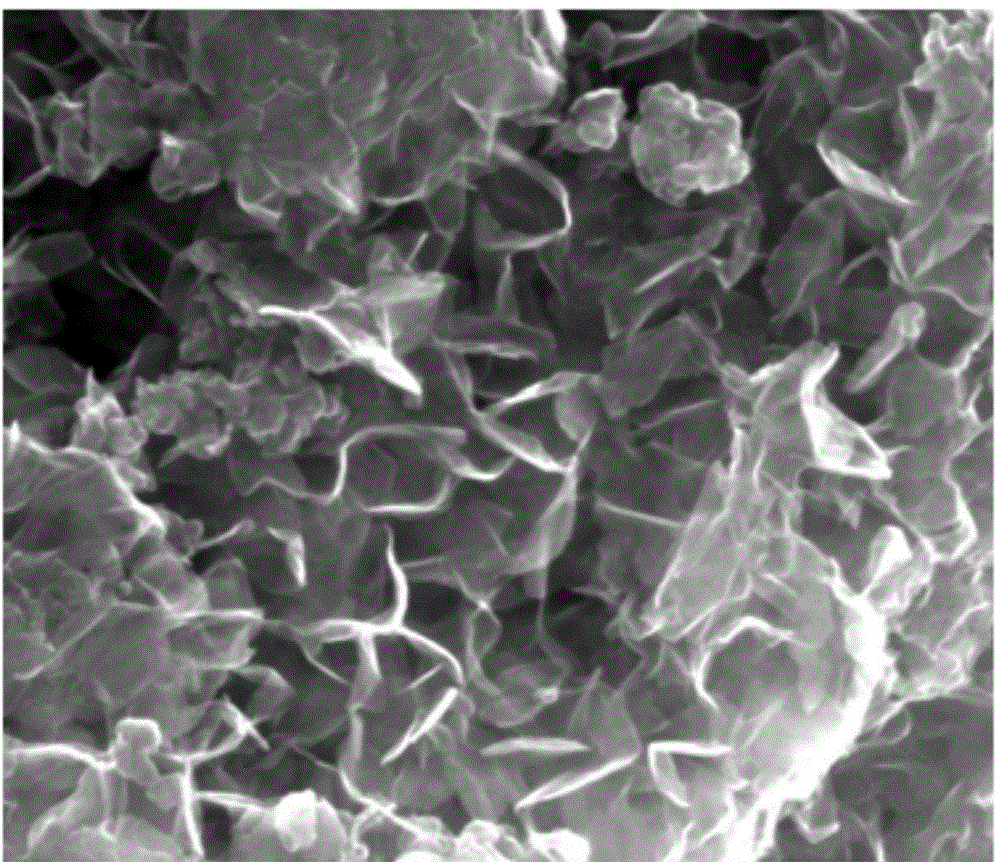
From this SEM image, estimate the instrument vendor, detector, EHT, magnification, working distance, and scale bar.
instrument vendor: FEI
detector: SE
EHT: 20 kV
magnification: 100 K X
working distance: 9.8 mm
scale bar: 500 nm